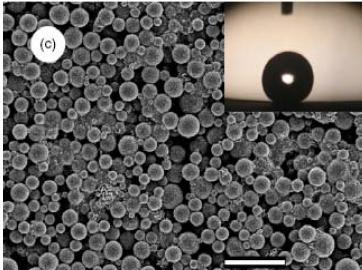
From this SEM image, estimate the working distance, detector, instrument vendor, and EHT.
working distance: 7 mm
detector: SEI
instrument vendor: JEOL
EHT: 3 kV